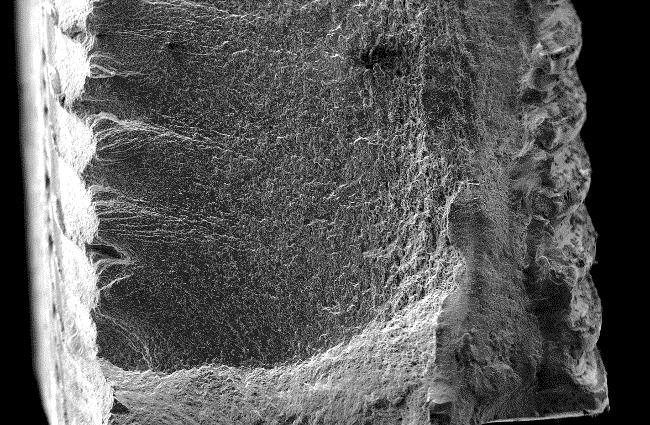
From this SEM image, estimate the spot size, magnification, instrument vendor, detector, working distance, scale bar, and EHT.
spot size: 3.5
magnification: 0.04 K X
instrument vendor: FEI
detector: SE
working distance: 6.7 mm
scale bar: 500000 nm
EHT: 10 kV